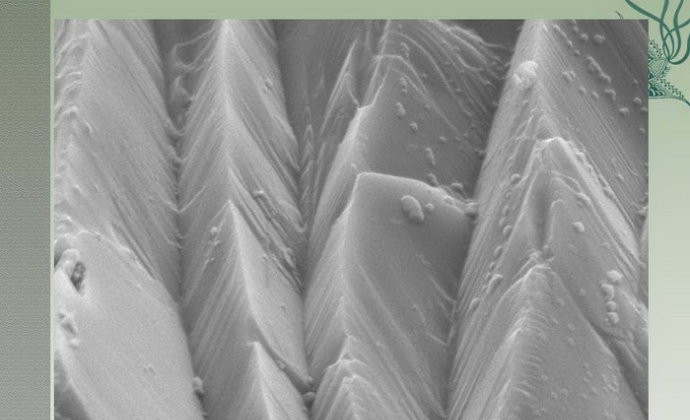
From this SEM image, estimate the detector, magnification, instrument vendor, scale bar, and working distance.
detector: SE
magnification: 3.5 K X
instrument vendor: Hitachi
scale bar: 10000 nm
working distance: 15 mm